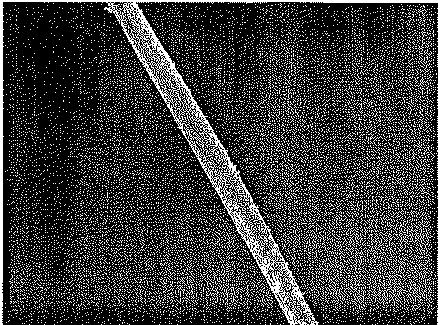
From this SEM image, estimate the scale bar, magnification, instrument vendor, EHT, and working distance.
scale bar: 10000 nm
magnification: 0.6 K X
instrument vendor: JEOL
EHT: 5 kV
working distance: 7 mm